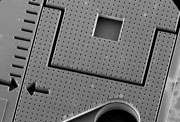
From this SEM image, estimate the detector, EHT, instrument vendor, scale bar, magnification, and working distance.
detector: SE(U)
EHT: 25 kV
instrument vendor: Hitachi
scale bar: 1000000 nm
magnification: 0.035 K X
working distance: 5.9 mm